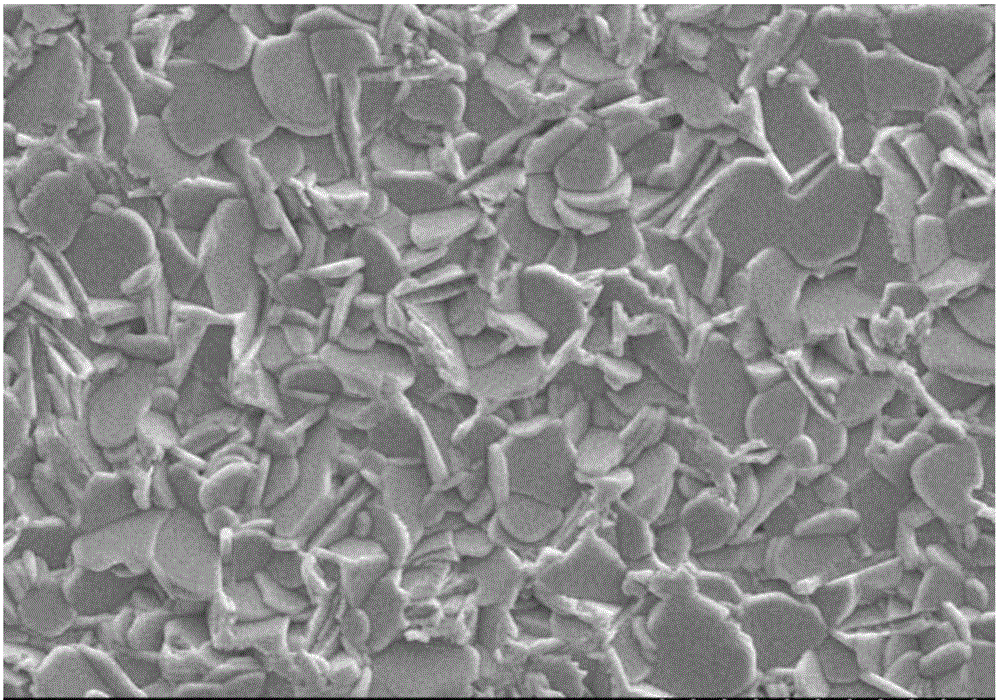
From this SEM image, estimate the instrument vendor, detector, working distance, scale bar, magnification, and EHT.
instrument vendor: Hitachi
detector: SE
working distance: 4 mm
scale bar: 20000 nm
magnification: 2 K X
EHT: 15 kV